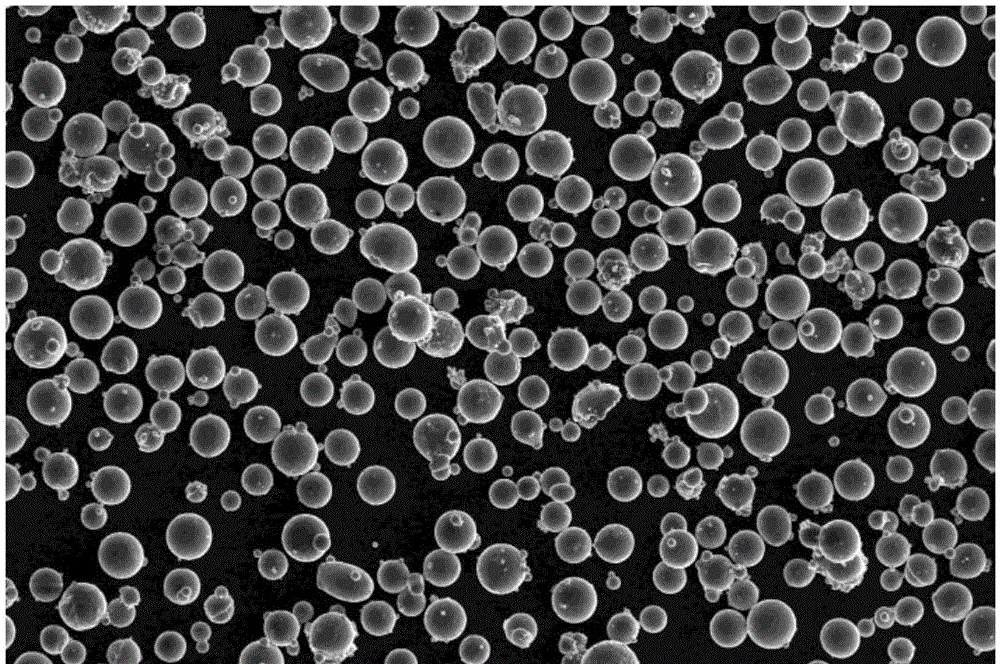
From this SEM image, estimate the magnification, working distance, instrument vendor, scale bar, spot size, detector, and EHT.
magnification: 0.5 K X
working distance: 15.6 mm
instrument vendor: FEI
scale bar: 300000 nm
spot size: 5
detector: SE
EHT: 20 kV